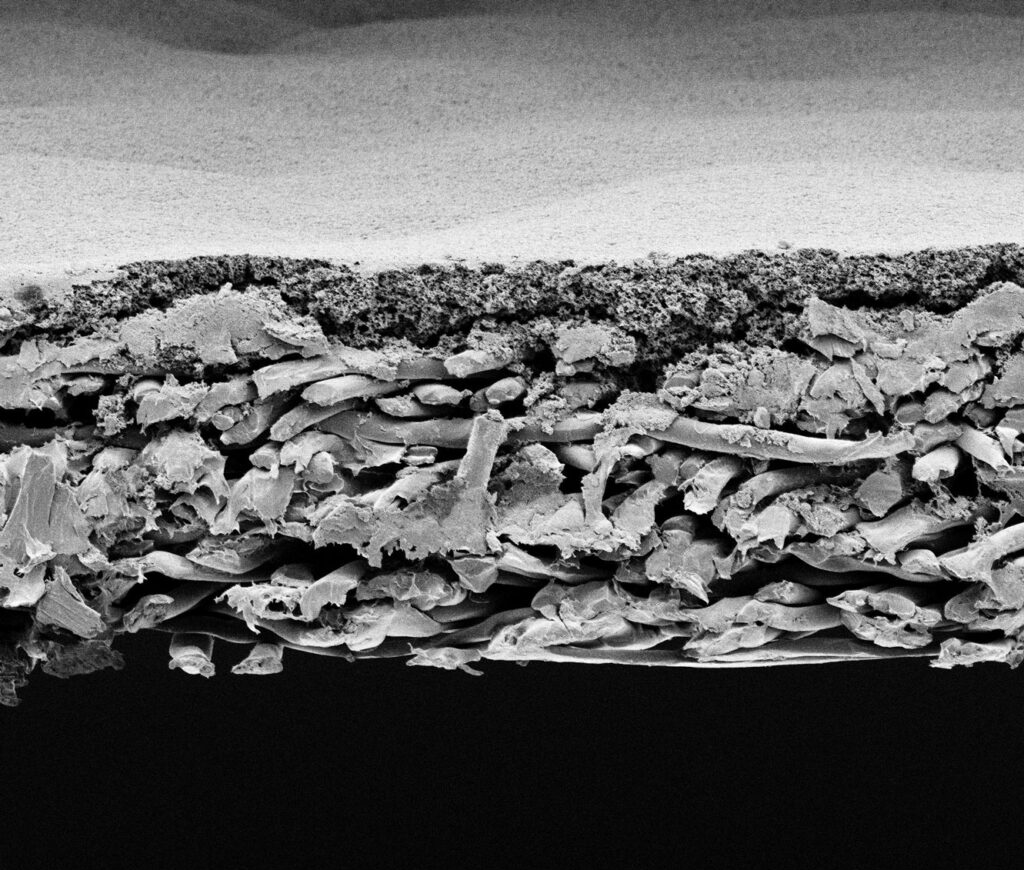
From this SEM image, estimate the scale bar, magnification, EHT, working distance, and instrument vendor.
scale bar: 50000 nm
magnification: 0.5 K X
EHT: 3 kV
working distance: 3.5 mm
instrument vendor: FEI